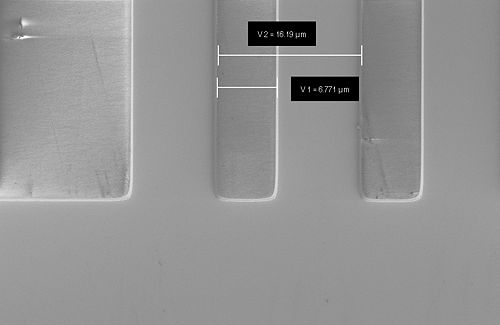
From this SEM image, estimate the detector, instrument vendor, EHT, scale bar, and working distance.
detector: SE2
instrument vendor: Zeiss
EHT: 2 kV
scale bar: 2000 nm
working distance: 8.6 mm